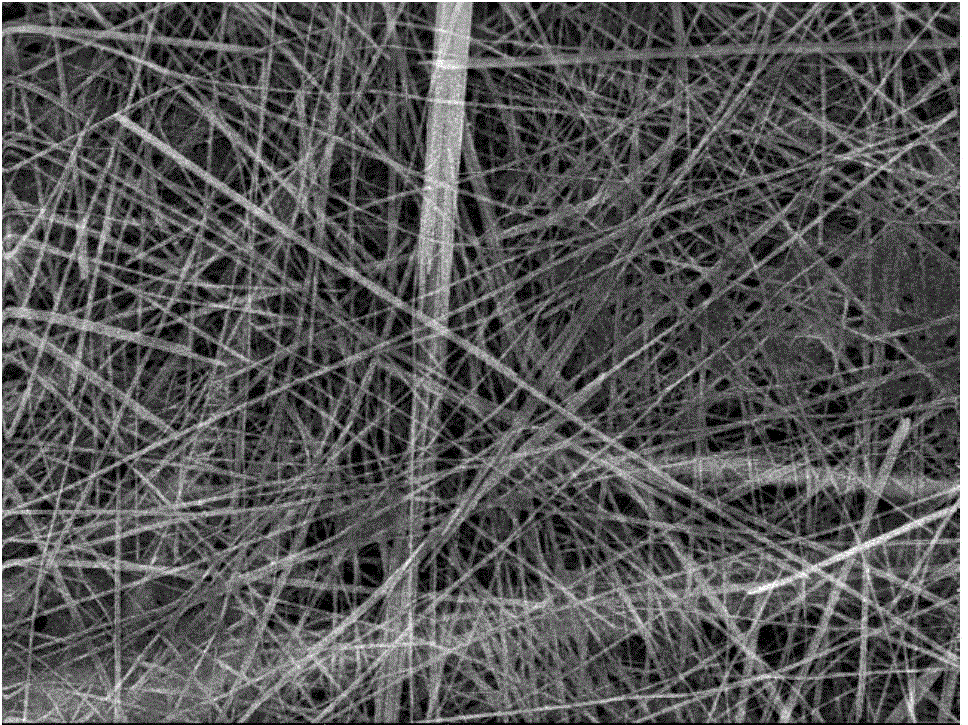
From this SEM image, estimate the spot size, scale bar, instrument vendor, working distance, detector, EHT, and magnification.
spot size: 4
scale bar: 2000 nm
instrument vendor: FEI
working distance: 3.9 mm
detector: TLD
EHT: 10 kV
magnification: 8 K X